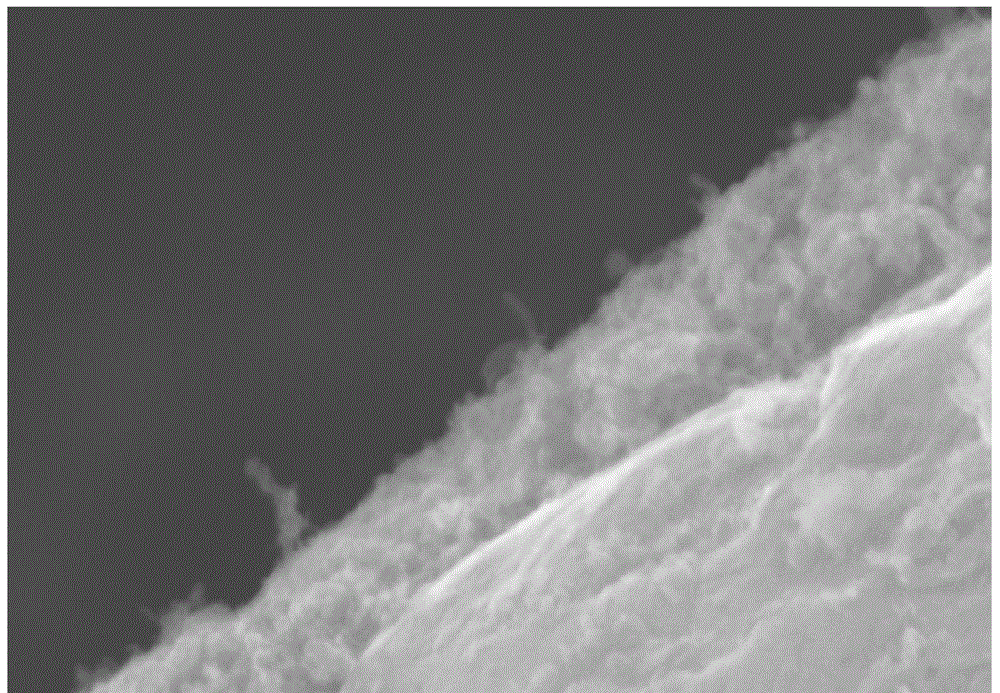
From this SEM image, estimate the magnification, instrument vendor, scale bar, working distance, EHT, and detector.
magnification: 50 K X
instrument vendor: Hitachi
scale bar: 1000 nm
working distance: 18.6 mm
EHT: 15 kV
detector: SE(M)